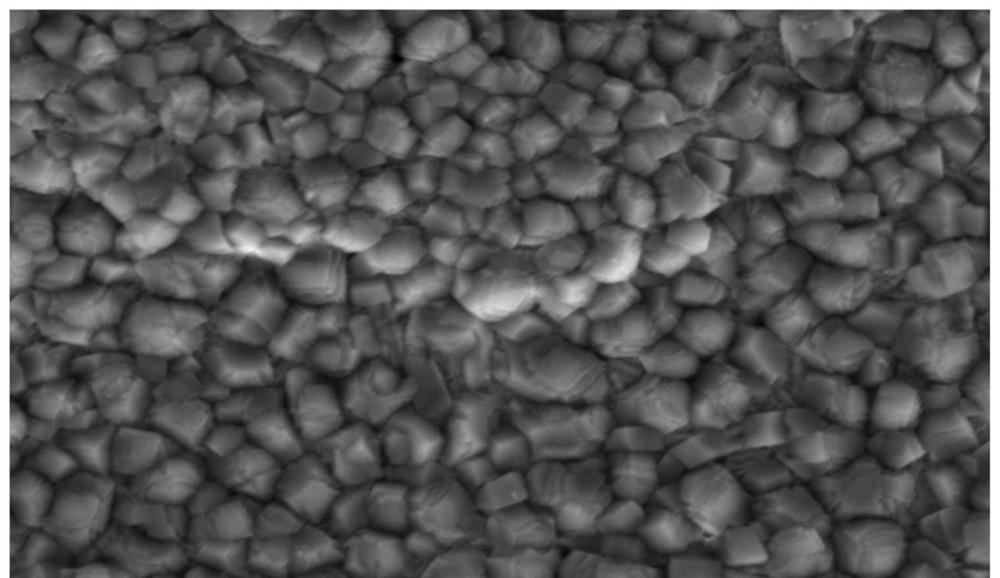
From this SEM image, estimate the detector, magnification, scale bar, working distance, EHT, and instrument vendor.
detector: SE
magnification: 3 K X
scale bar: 10000 nm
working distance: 9.6 mm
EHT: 15 kV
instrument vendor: Hitachi